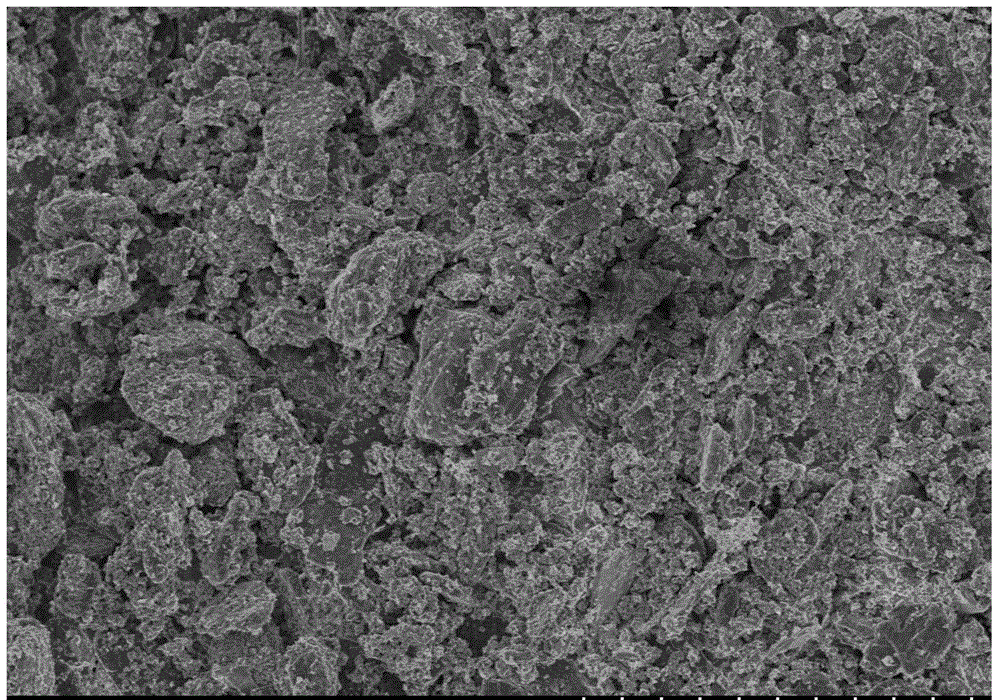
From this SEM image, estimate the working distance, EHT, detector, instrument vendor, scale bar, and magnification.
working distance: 8.1 mm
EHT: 3 kV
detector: SE(M)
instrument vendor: Hitachi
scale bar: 50000 nm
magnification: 1 K X